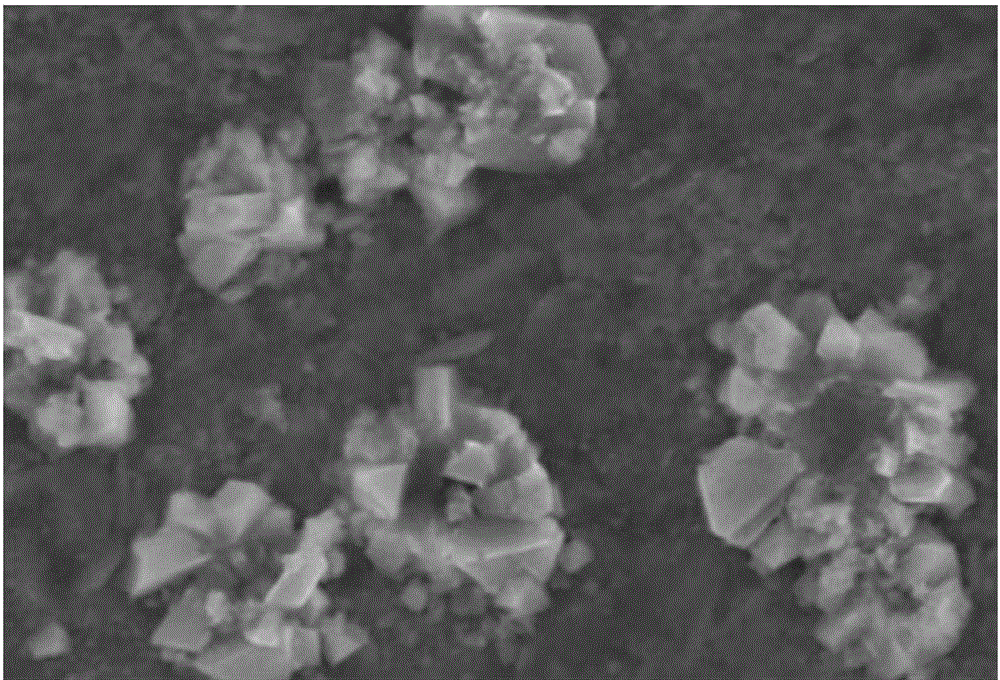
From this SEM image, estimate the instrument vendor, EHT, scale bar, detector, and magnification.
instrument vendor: JEOL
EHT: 20 kV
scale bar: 5000 nm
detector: SEI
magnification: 5 K X